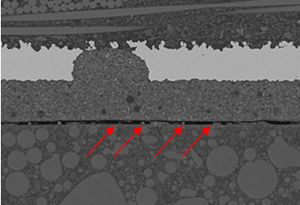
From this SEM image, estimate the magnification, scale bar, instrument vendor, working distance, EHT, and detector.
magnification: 0.75 K X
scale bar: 50000 nm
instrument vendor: Hitachi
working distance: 10.1 mm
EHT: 15 kV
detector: BSE3D